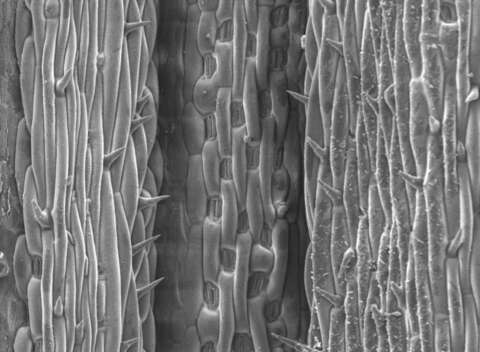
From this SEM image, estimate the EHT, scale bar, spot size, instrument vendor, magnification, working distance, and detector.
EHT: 10 kV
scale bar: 100000 nm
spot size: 3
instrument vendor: FEI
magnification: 0.2 K X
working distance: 10.1 mm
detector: SE